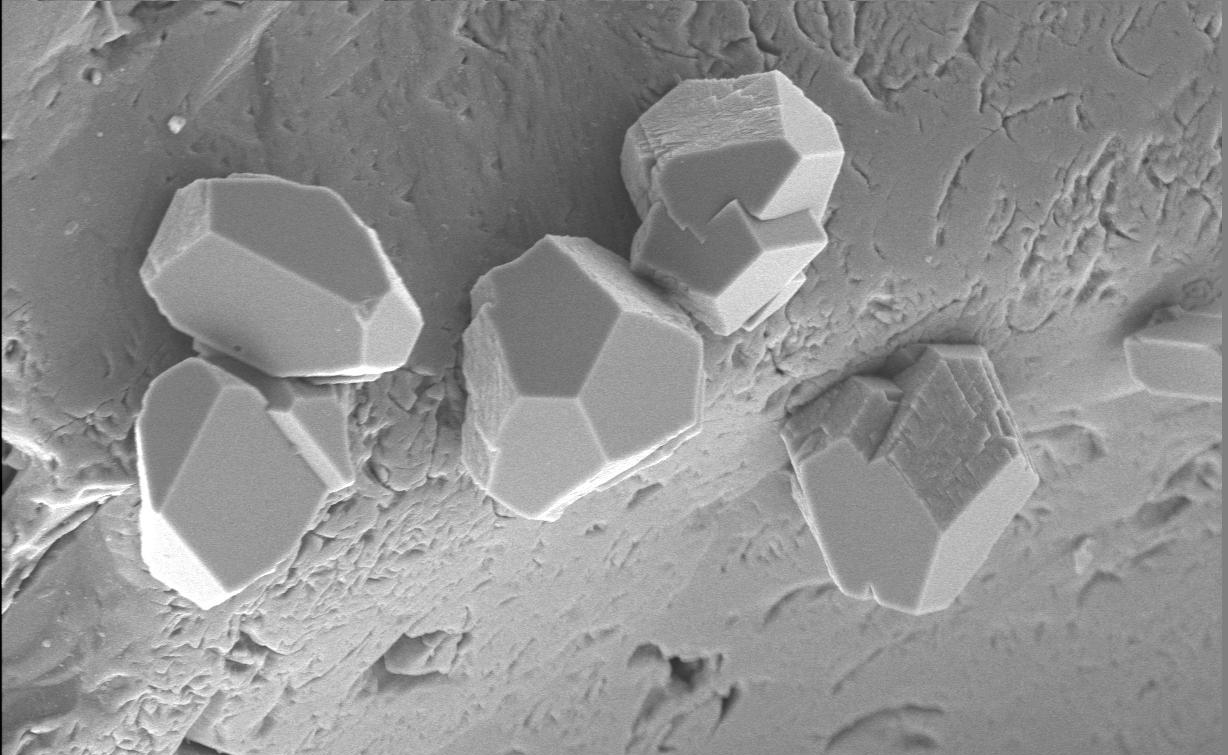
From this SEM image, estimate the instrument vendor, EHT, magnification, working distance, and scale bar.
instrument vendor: JEOL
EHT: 9 kV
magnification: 1.5 K X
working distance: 11 mm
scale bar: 10000 nm